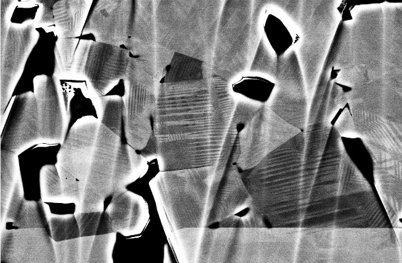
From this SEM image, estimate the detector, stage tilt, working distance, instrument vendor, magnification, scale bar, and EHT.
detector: CBS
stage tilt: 0°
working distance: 6 mm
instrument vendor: FEI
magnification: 20 K X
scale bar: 5000 nm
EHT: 10 kV